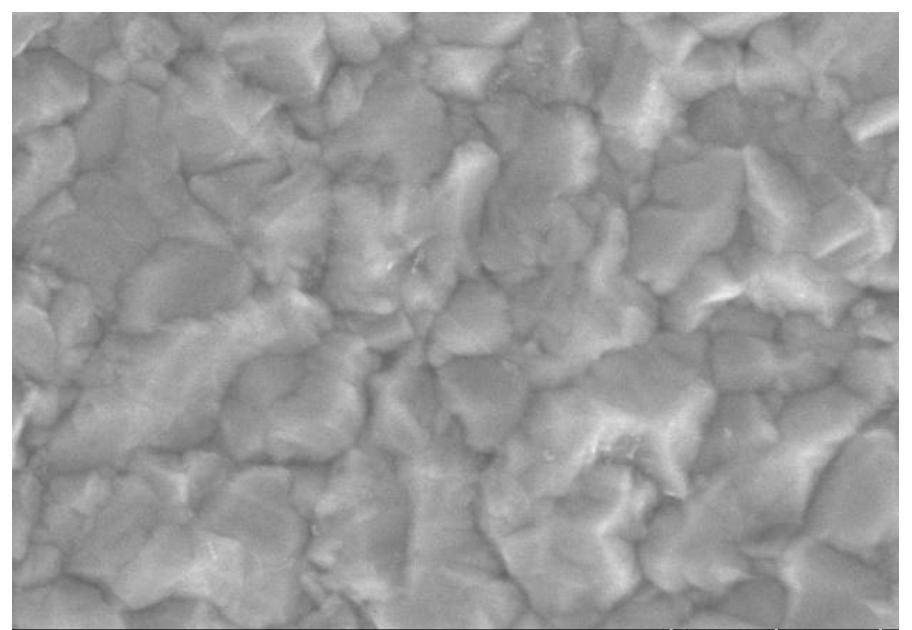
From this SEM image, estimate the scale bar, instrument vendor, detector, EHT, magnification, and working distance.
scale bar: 500 nm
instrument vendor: Hitachi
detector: SE(UL)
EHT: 10 kV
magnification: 60 K X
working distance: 8.4 mm